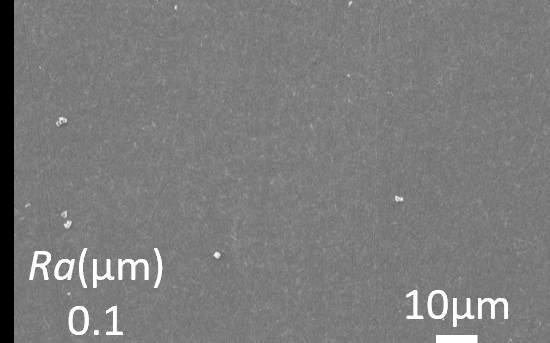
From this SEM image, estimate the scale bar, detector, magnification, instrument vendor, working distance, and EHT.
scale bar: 10000 nm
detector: SED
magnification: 1 K X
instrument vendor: JEOL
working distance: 11.6 mm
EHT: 10 kV